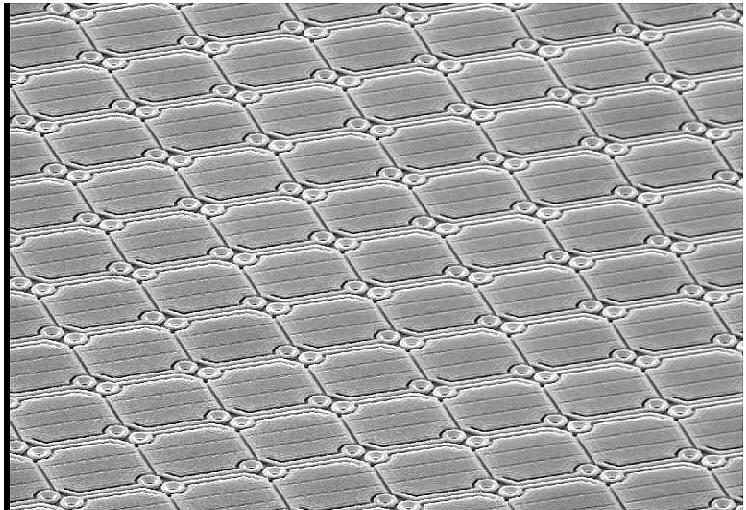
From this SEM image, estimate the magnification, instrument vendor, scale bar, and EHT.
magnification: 0.5 K X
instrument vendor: JEOL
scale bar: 60000 nm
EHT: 15 kV